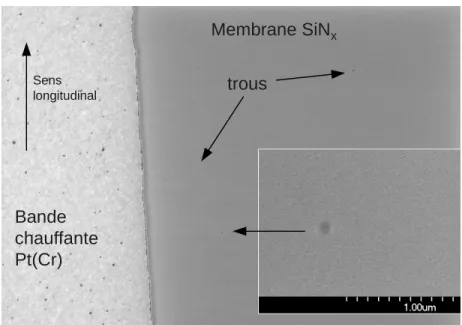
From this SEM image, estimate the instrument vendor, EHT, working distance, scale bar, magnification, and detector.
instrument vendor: Hitachi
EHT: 2 kV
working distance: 4.3 mm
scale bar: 5000 nm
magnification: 8 K X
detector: SE(U)